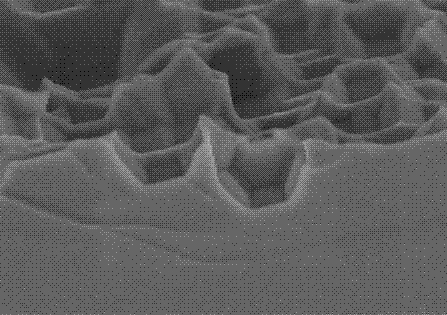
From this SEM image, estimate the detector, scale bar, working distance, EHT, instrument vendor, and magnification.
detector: SE(M,LA3)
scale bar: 1000 nm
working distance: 7.5 mm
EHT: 3 kV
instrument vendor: Hitachi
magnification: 30 K X